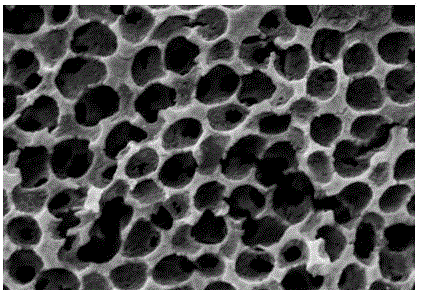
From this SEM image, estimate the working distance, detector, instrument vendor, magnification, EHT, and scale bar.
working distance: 7.4 mm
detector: SE(M)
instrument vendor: Hitachi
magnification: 35 K X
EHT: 5 kV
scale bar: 1000 nm